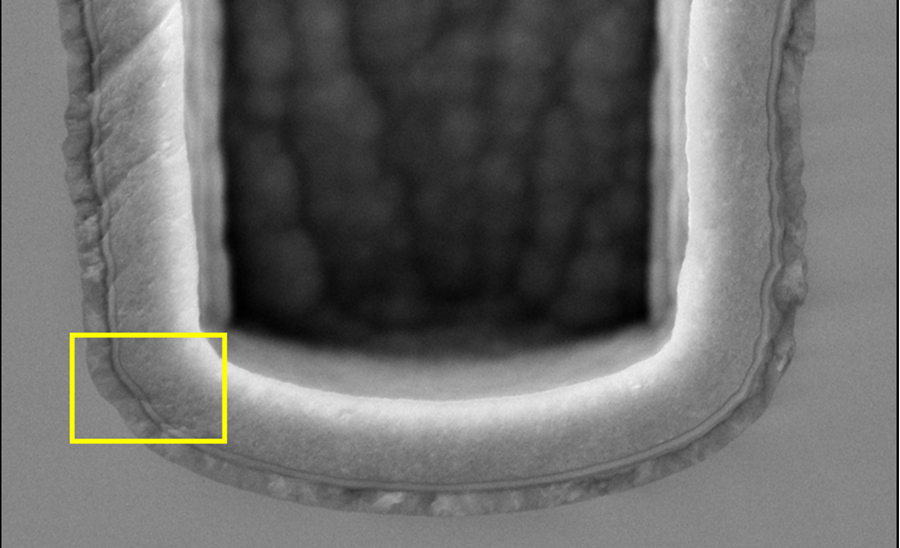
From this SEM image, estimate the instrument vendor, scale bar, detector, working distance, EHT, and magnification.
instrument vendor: Hitachi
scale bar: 2000 nm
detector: SE(U,LA20)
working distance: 2 mm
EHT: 5 kV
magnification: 25 K X